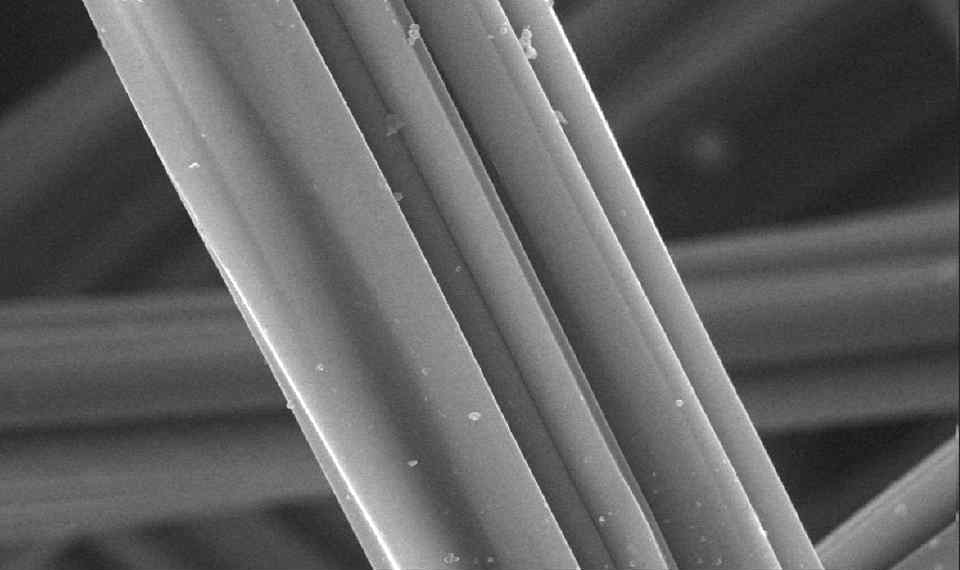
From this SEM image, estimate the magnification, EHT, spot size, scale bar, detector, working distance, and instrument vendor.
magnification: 3 K X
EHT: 15 kV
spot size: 3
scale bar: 10000 nm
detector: GSE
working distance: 9.9 mm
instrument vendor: FEI